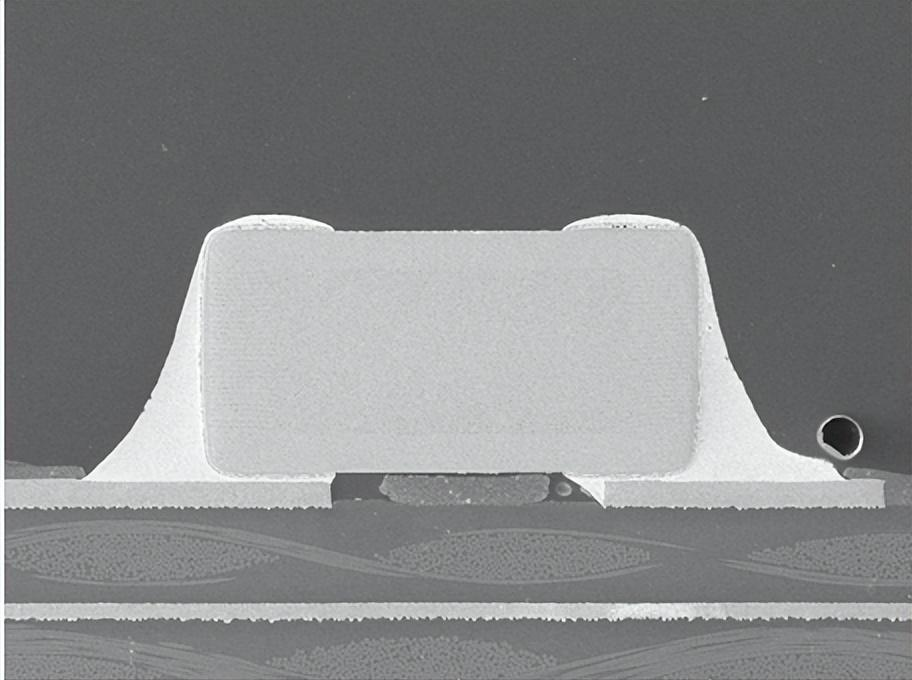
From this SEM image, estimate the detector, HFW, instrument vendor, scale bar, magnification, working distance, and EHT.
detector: SE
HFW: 1810 µm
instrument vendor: Tescan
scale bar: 500000 nm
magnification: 0.153 K X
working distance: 15.09 mm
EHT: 20 kV